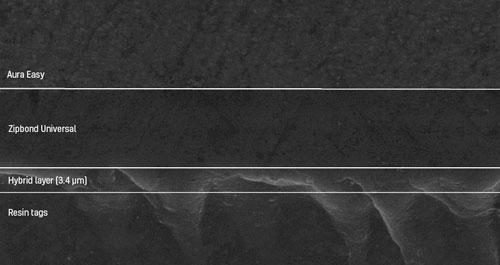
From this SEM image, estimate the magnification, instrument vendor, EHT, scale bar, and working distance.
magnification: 2 K X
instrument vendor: JEOL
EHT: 15 kV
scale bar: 10000 nm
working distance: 21 mm